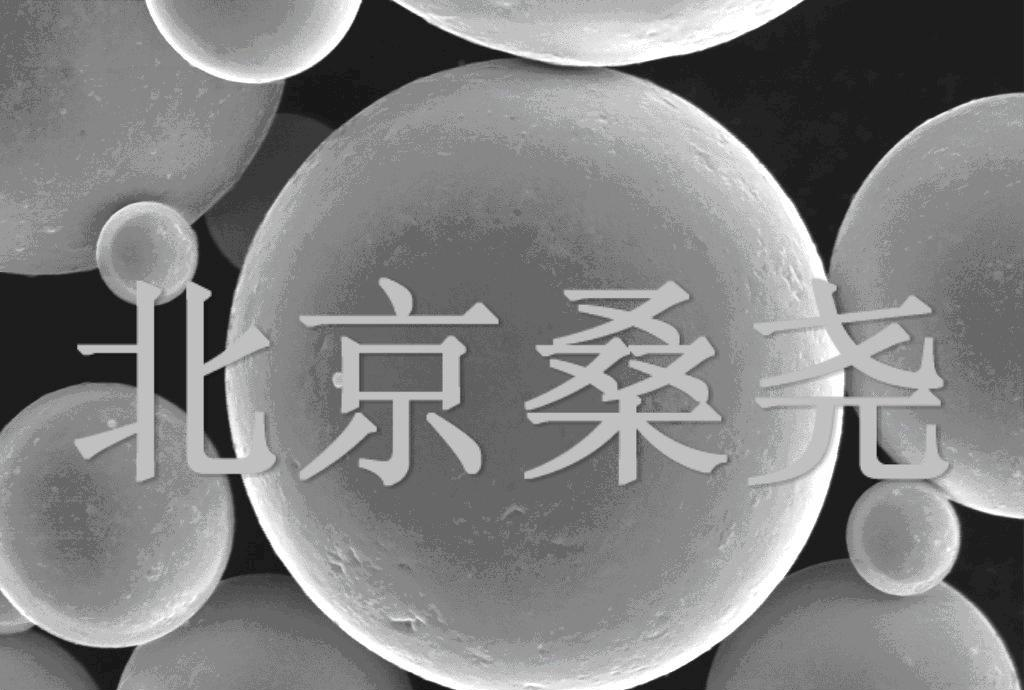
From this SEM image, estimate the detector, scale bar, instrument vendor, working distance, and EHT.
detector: SE1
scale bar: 2000 nm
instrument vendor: Zeiss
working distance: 19 mm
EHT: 20 kV